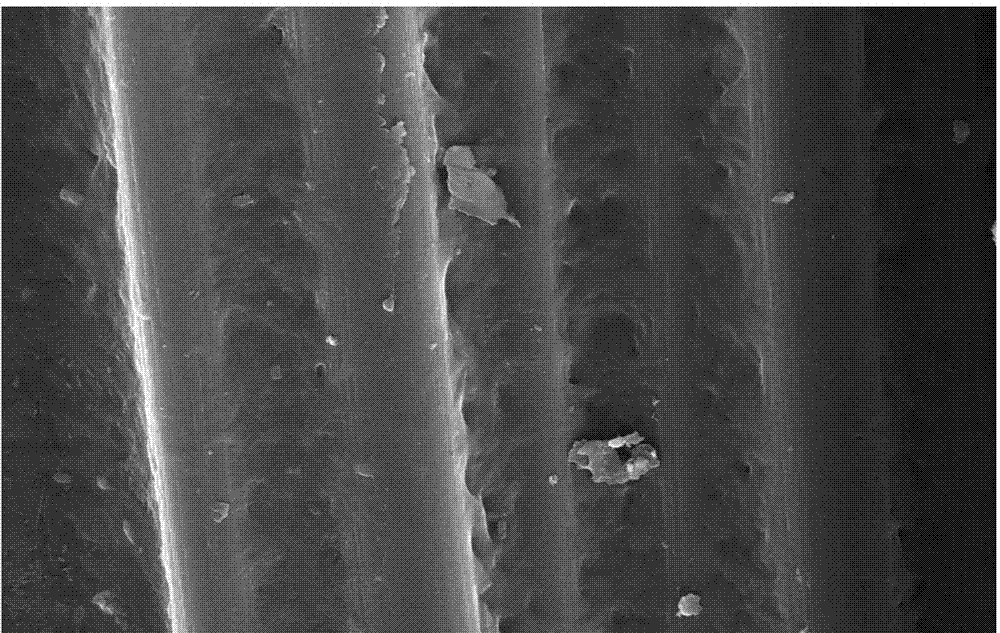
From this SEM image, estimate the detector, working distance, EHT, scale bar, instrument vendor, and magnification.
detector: ETD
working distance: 5.2 mm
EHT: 15 kV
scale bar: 20000 nm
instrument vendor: FEI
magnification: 8 K X